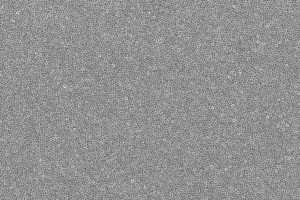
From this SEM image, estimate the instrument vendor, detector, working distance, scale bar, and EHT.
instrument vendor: Zeiss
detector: InLens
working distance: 9.7 mm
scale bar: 5000 nm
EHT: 5 kV